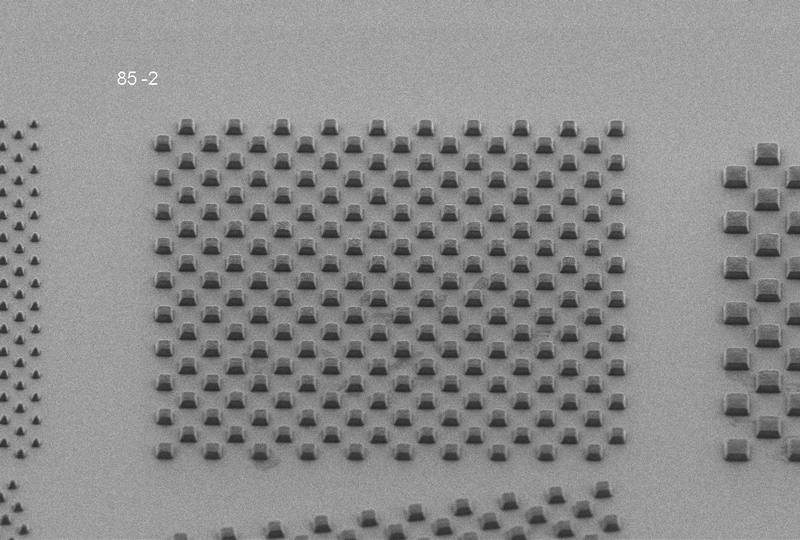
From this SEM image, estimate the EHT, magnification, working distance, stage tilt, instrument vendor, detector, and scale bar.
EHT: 1.5 kV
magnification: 1.16 K X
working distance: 7.3 mm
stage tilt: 45°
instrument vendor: Zeiss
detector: HE-SE2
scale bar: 10000 nm